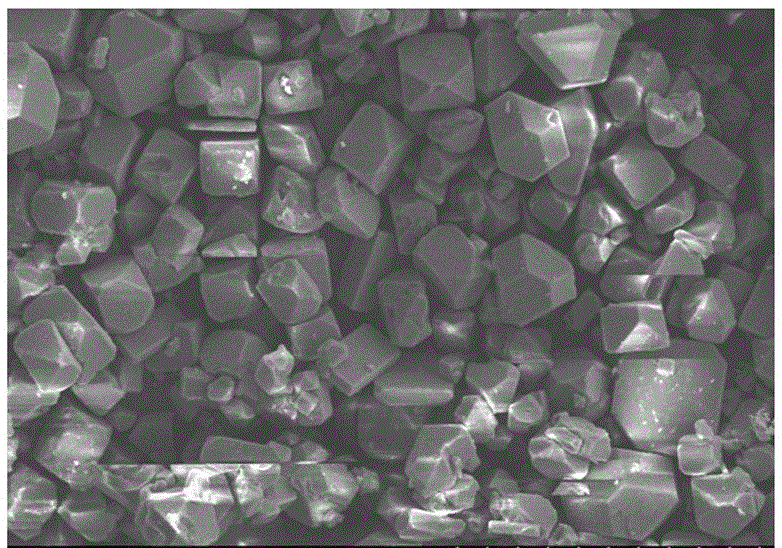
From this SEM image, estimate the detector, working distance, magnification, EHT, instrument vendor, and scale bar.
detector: SE(M)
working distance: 13.8 mm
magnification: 0.5 K X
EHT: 15 kV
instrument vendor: Hitachi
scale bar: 100000 nm